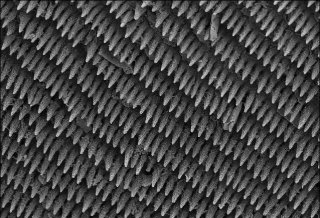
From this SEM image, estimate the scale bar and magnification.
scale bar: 50000 nm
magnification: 0.5 K X